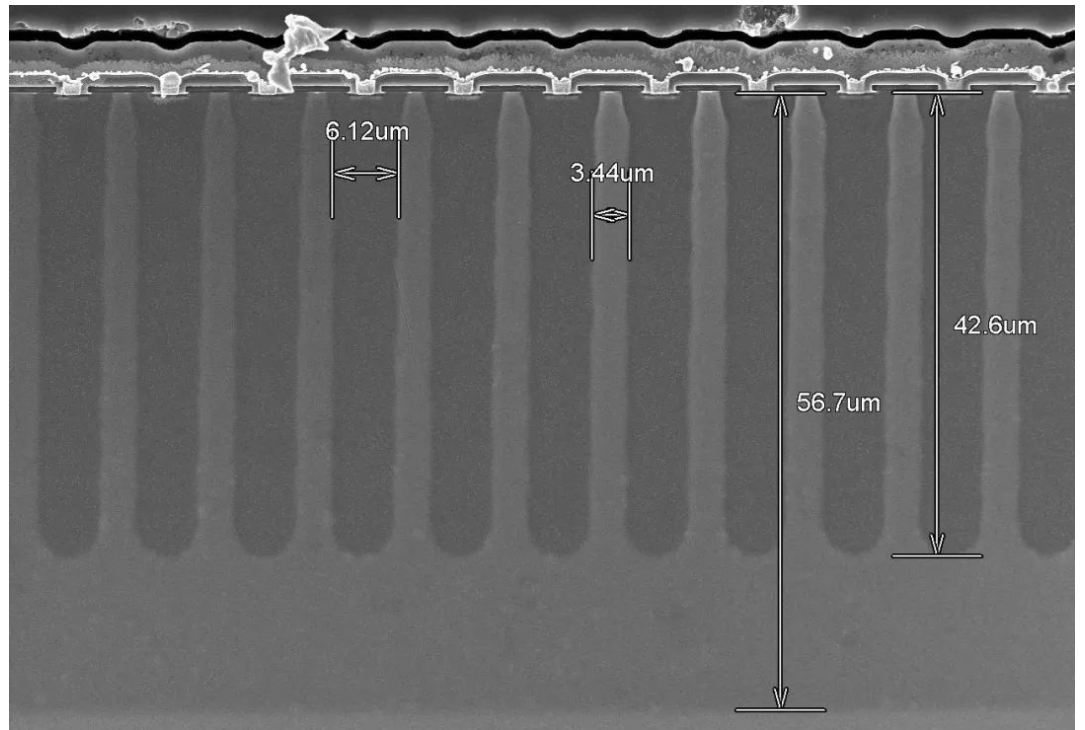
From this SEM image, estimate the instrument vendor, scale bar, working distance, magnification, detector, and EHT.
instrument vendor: Hitachi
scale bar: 40000 nm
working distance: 7.9 mm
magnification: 1.3 K X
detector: SE(UL)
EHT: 10 kV